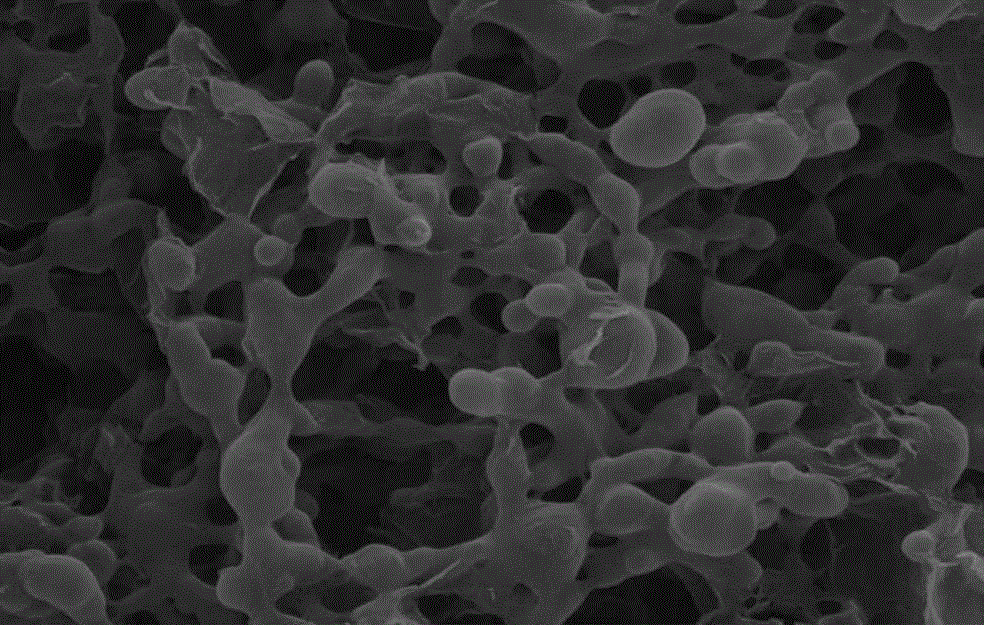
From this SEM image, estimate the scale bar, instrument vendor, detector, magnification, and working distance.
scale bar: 2000 nm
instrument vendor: Tescan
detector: SE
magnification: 30 K X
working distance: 11.79 mm